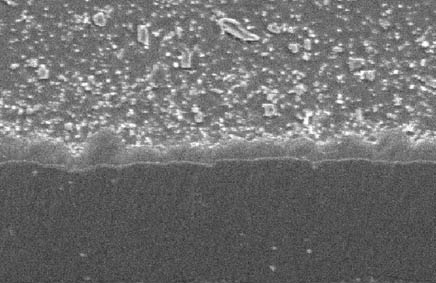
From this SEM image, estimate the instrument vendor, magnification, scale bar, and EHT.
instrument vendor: JEOL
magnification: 5 K X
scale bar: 5000 nm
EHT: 10 kV